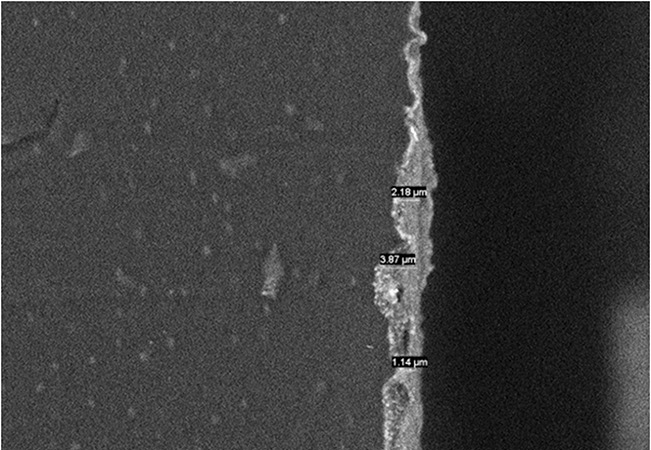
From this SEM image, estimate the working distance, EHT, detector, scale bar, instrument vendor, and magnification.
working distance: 9.5 mm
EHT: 10 kV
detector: BSE3D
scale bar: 20000 nm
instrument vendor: Hitachi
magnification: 2 K X